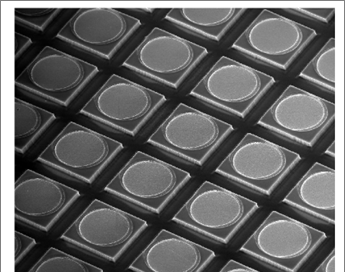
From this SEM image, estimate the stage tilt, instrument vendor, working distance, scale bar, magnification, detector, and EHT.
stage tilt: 0°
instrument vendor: FEI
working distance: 4 mm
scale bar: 40000 nm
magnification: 1.231 K X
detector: TLD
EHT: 10 kV